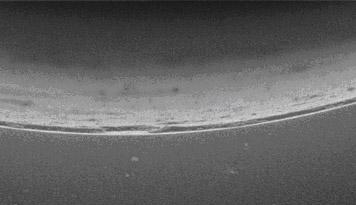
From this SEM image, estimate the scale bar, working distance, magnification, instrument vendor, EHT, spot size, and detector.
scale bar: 1000 nm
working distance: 6.8 mm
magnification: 20 K X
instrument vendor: FEI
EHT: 22 kV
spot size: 2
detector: SE